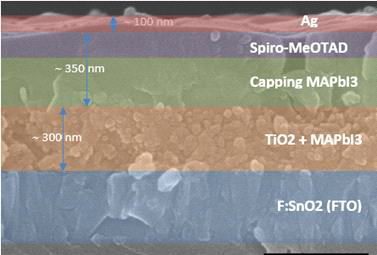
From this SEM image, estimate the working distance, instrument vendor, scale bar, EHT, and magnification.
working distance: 12.6 mm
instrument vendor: Hitachi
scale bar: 500 nm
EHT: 15 kV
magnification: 70 K X